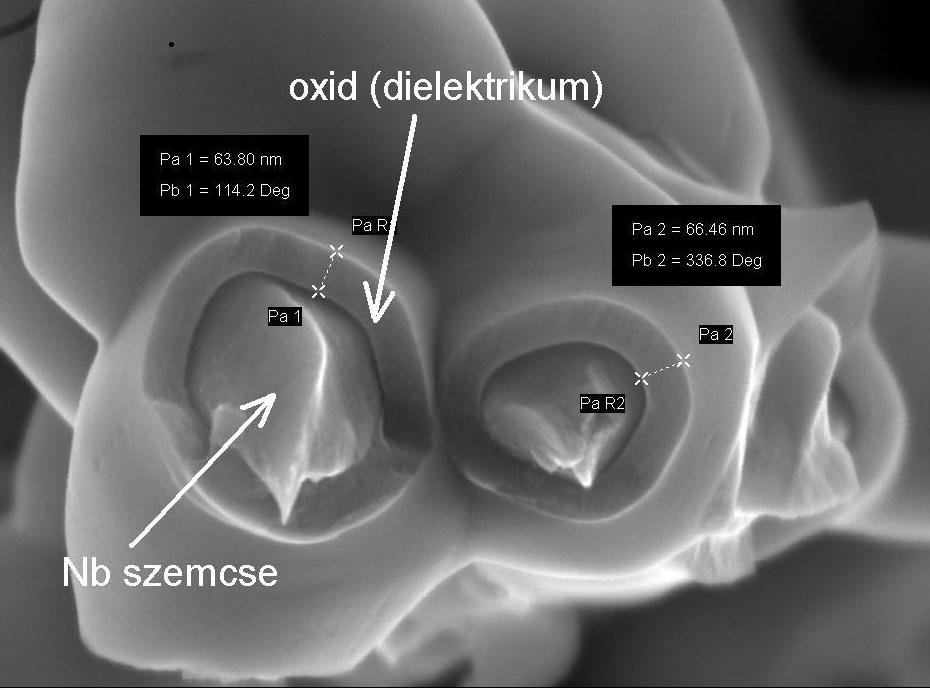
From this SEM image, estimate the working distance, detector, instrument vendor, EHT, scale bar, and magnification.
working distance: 3 mm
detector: InLens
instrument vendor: Zeiss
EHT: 10 kV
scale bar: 100 nm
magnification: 180 K X